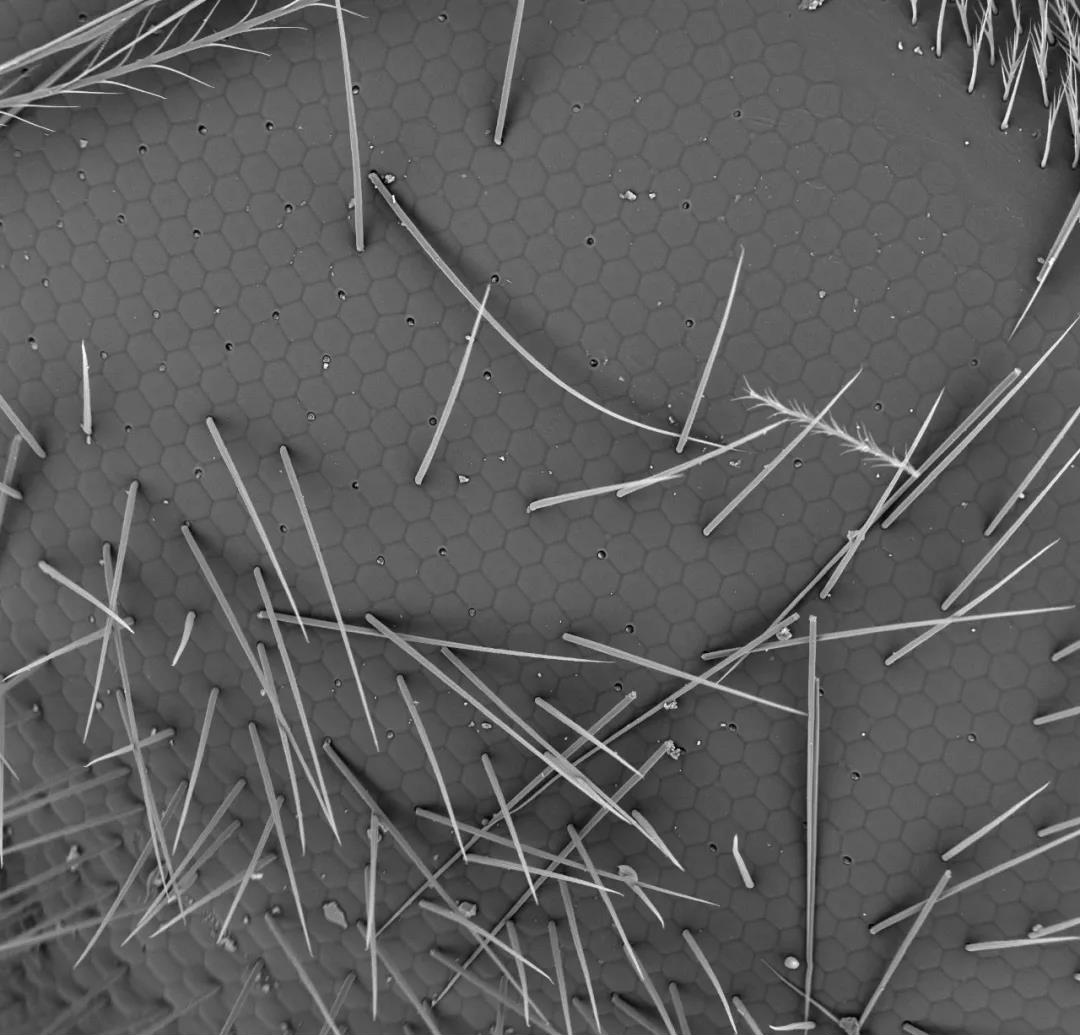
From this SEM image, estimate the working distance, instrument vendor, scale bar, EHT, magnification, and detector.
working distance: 5.7 mm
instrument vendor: other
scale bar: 100000 nm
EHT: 15 kV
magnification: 0.5 K X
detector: BSE